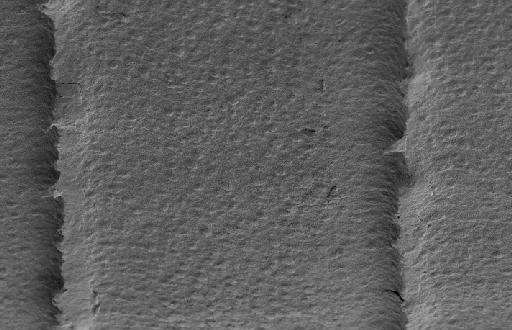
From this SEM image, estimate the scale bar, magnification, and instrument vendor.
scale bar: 10000 nm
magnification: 5 K X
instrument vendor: FEI